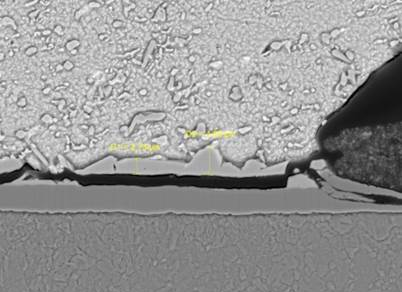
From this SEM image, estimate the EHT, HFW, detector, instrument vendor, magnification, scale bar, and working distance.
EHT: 20 kV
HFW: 69.2 µm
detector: BSE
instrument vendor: Tescan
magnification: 4 K X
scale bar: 20000 nm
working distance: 11.99 mm